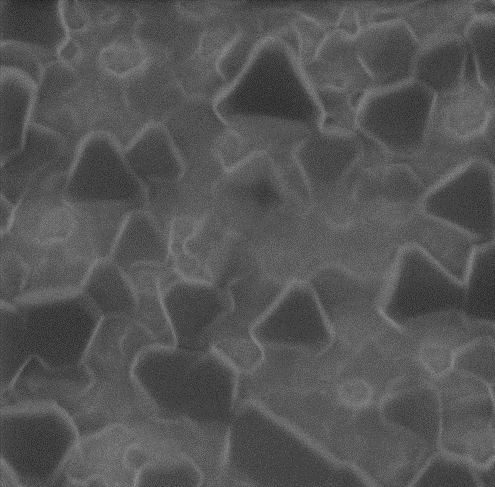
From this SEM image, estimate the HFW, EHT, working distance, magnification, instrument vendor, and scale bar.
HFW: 1.3 µm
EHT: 25 kV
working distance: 14.4 mm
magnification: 87.9231 K X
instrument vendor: Zeiss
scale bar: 100 nm